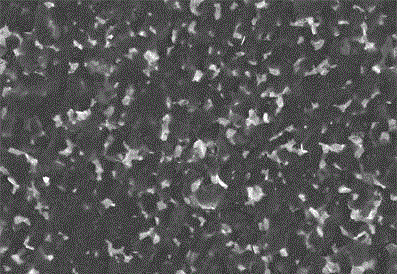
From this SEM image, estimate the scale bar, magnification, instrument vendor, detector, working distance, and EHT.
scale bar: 20000 nm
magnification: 2 K X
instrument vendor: Hitachi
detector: SE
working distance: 13.2 mm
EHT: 15 kV